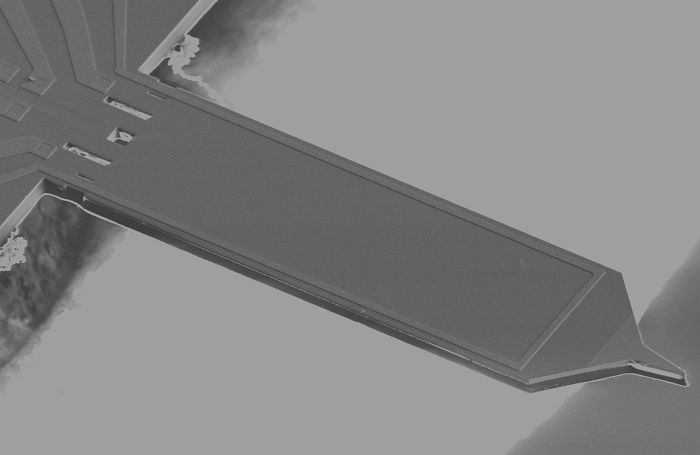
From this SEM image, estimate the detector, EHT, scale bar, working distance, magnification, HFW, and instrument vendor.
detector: ETD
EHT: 5 kV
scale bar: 100000 nm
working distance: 4 mm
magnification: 0.326 K X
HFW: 635 µm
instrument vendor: FEI